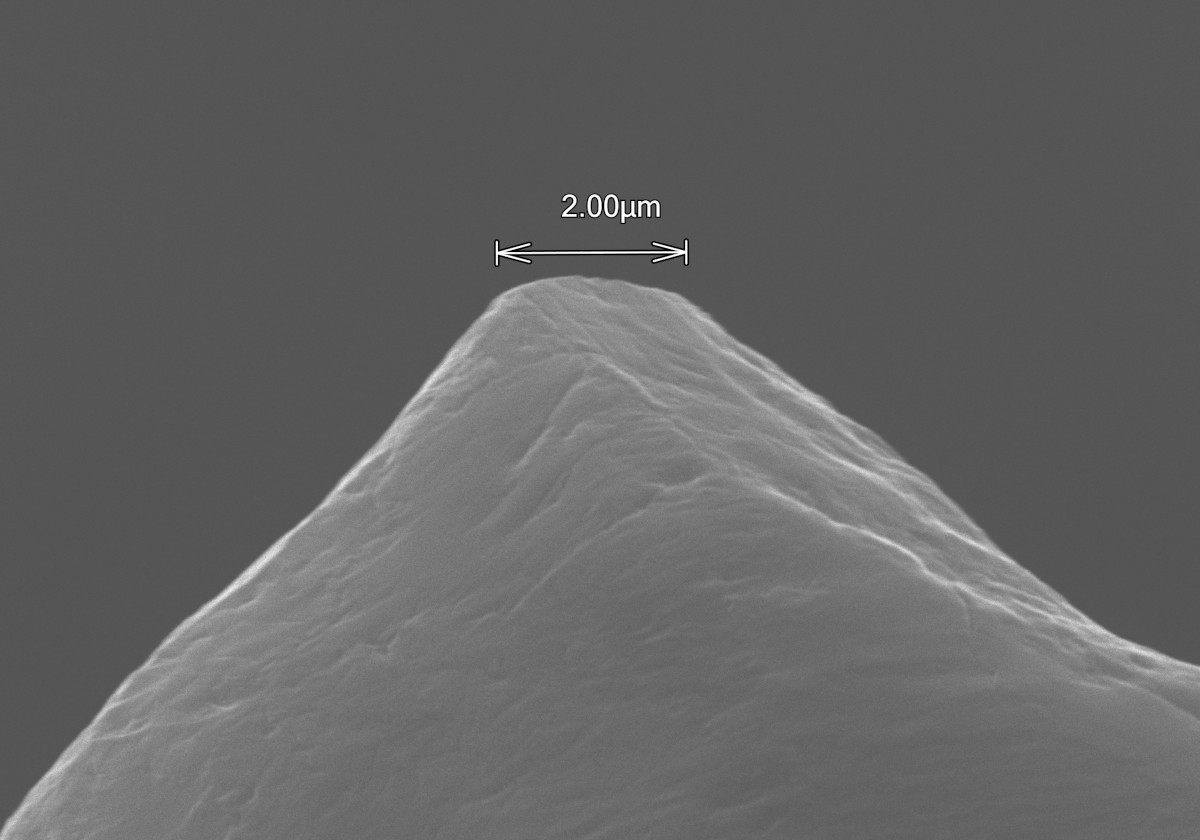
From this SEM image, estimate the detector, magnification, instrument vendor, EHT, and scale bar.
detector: SE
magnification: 10 K X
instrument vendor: Hitachi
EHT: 20 kV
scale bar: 5000 nm